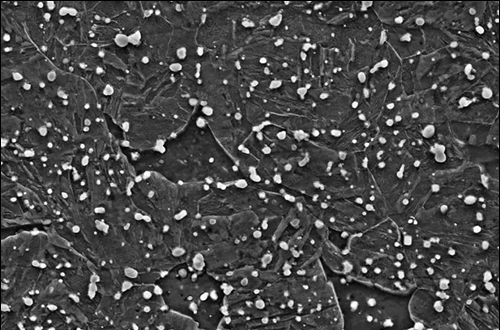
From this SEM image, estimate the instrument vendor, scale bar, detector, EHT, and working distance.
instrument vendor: Zeiss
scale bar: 2000 nm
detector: SE2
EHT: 15 kV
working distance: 10.4 mm